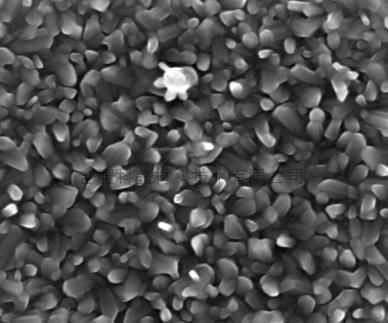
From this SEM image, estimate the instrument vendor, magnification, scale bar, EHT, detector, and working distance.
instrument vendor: FEI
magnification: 40 K X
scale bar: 1000 nm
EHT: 20 kV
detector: TLD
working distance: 6 mm